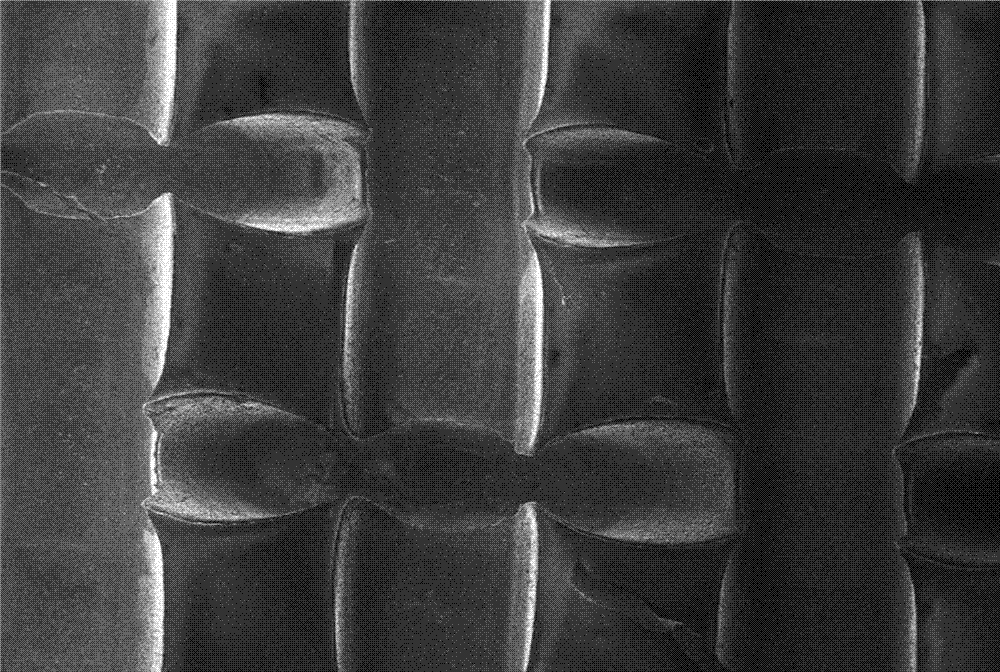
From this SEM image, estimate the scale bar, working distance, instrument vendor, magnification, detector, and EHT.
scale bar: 100000 nm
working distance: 13.5 mm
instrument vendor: Zeiss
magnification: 0.121 K X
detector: SE1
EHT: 2 kV